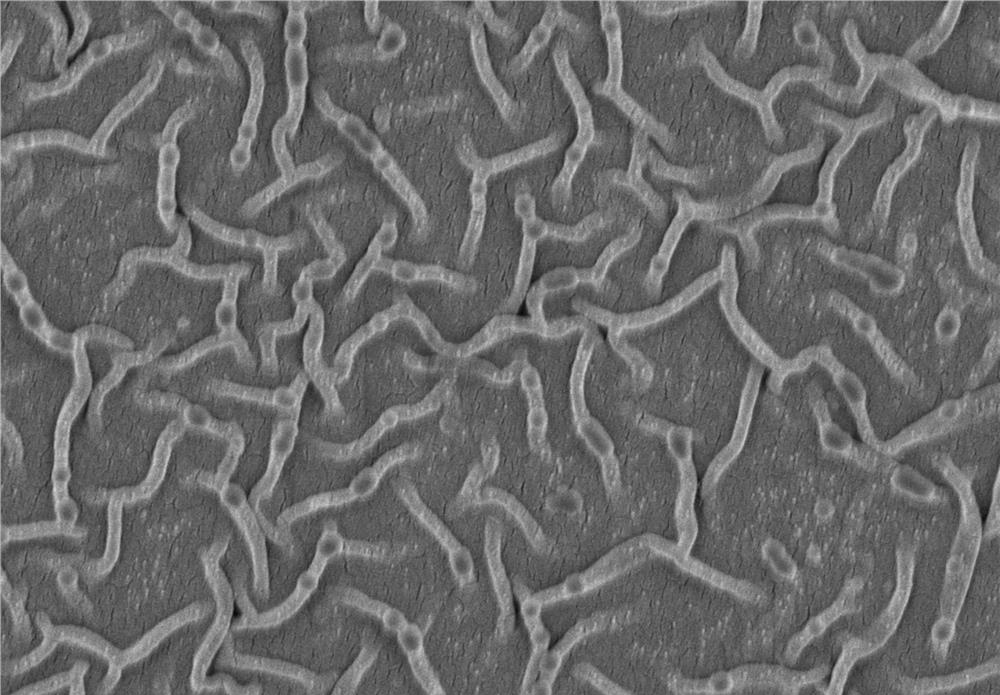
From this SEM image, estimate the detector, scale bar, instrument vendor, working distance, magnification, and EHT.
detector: SE(M)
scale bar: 2000 nm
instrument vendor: Hitachi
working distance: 6.4 mm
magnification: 20 K X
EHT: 3 kV